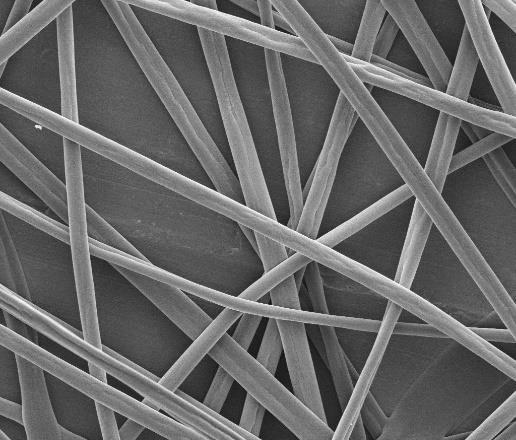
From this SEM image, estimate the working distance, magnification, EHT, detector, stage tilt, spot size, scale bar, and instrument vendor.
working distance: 11.9 mm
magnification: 5 K X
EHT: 5 kV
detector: ETD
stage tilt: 0°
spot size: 3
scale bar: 10000 nm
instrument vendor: FEI